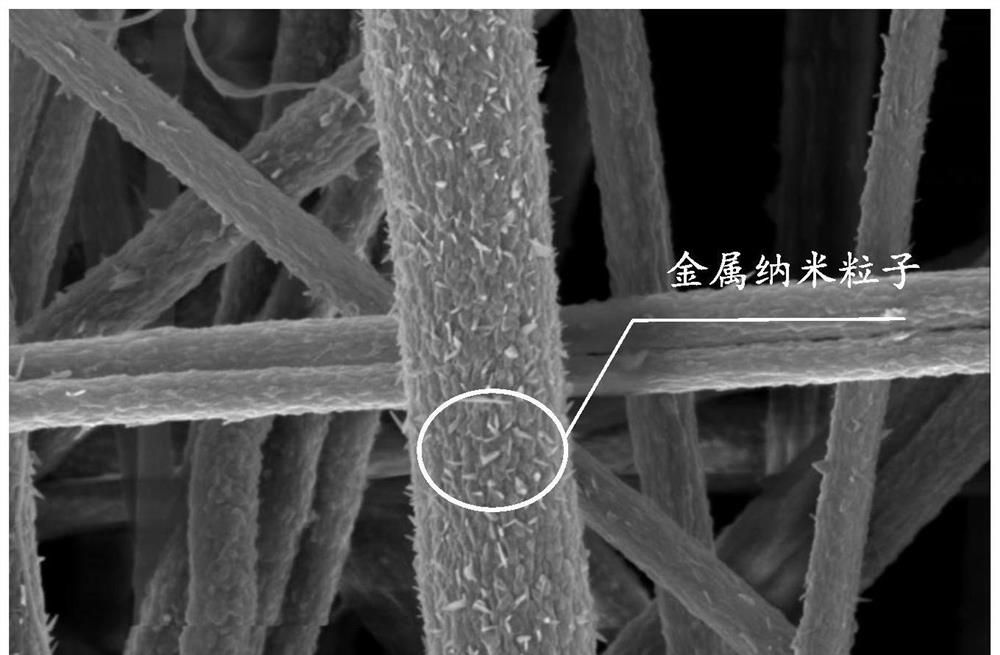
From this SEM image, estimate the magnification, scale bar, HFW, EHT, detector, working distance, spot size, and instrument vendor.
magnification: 40 K X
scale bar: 3000 nm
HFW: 10.4 µm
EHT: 5 kV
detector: ETD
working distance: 9.5 mm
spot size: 8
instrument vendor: FEI